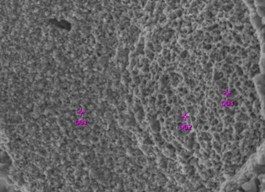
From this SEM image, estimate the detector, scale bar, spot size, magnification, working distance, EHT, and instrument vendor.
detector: SEI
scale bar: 10000 nm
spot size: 48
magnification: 2 K X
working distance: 10 mm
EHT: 20 kV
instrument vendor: JEOL